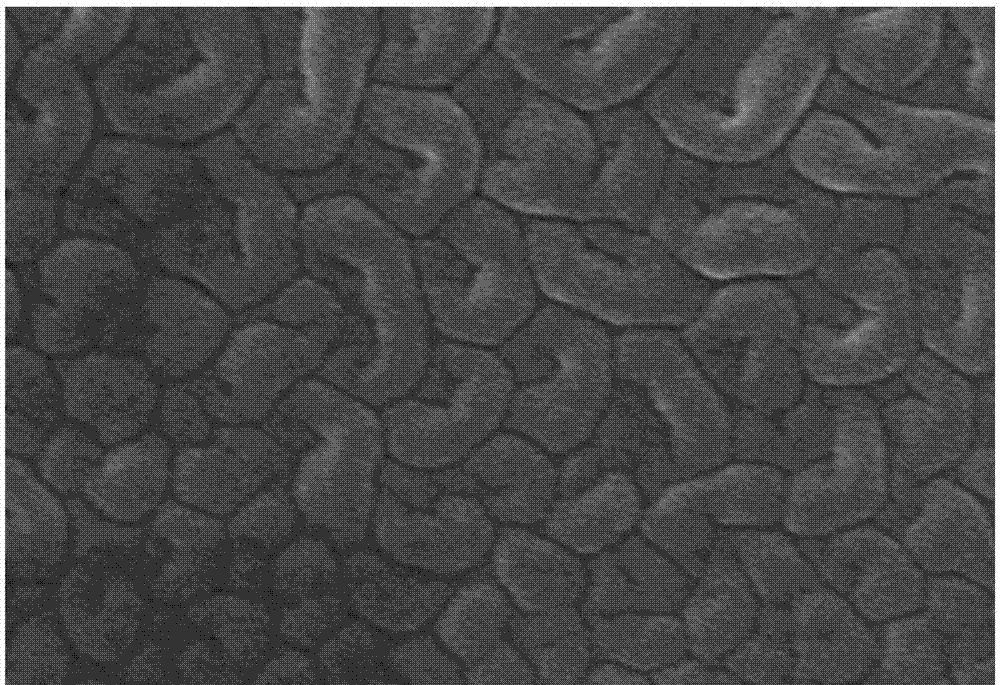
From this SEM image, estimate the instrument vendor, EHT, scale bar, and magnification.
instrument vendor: Hitachi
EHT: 15 kV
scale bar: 100 nm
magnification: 100 K X